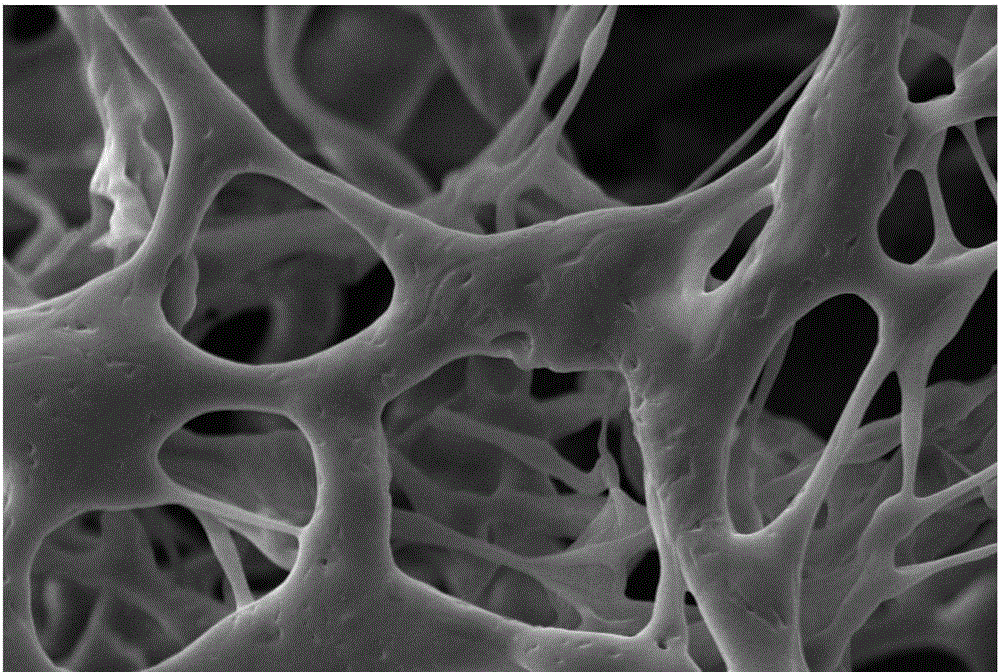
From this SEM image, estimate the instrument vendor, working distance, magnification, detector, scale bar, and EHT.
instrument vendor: Zeiss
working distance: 9 mm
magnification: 2.01 K X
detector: SE1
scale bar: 2000 nm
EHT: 10 kV